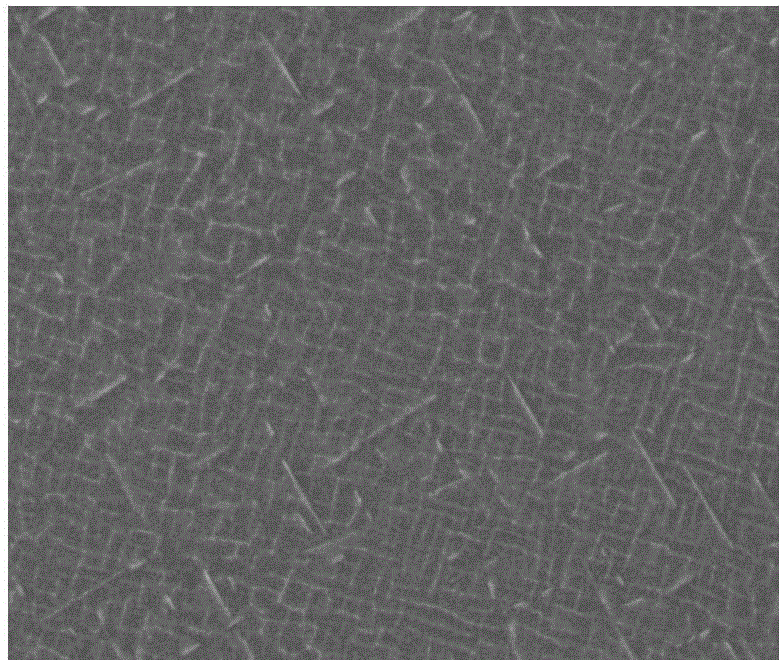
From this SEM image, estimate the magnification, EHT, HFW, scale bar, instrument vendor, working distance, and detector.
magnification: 10 K X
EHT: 30 kV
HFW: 29.8 µm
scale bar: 10000 nm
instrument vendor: FEI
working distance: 10.7 mm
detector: BSED